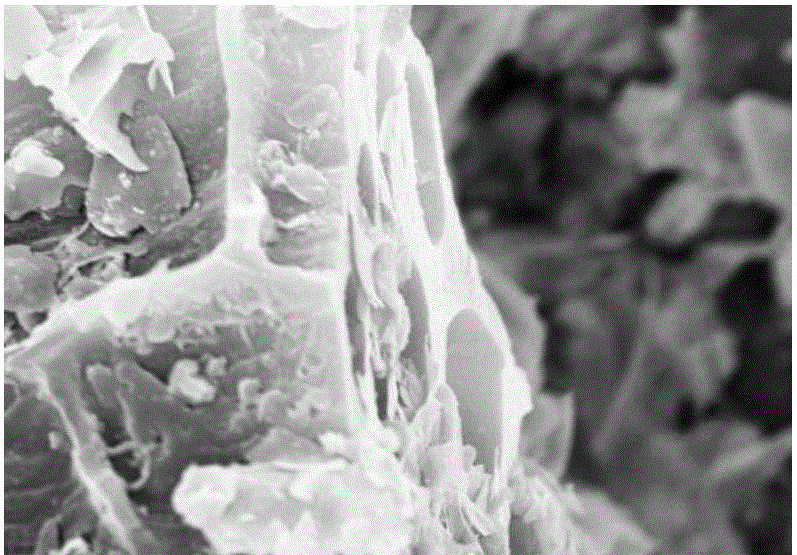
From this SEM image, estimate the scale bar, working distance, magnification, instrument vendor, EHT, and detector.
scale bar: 5000 nm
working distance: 7.1 mm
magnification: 10 K X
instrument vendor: Hitachi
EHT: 15 kV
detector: SE(M)